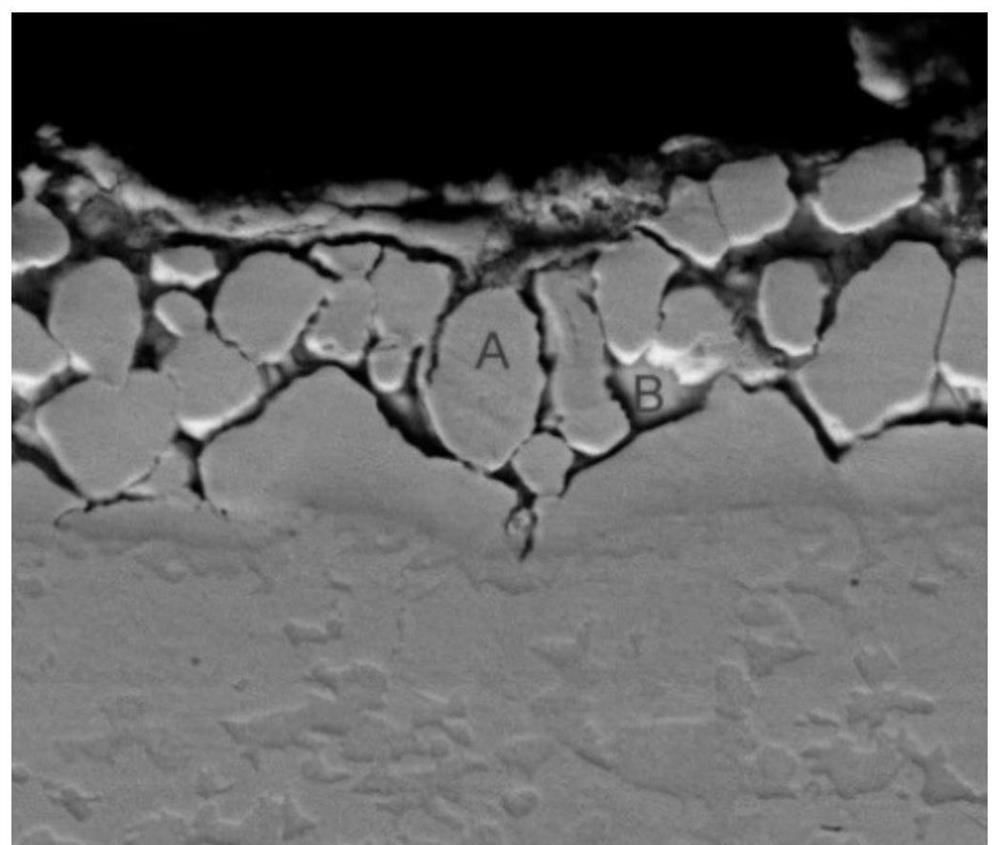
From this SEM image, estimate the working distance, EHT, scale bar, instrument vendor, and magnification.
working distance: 11.2 mm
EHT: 20 kV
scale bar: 30000 nm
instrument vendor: FEI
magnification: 5 K X